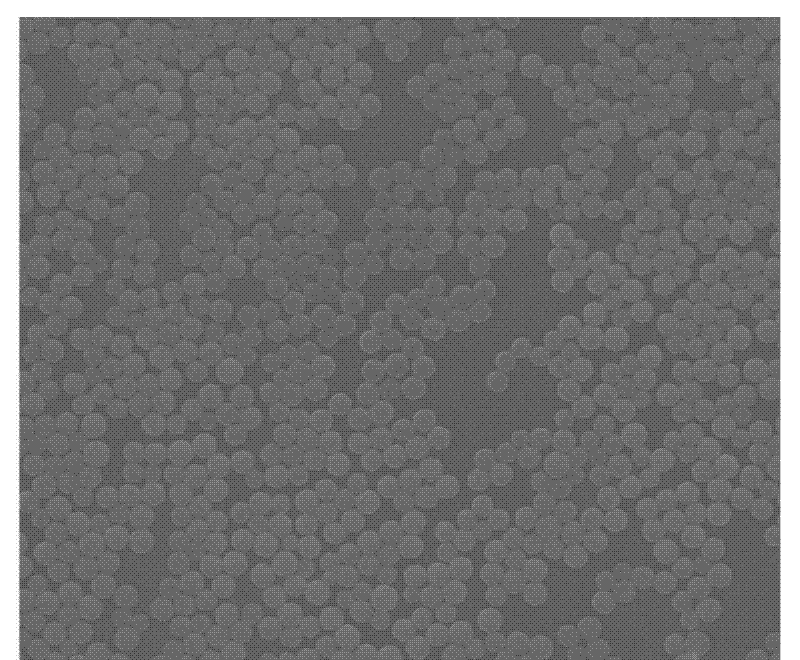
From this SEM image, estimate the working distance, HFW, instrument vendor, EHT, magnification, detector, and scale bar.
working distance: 8.1 mm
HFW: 33.8 µm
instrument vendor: FEI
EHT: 20 kV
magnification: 4 K X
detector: ETD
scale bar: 5000 nm